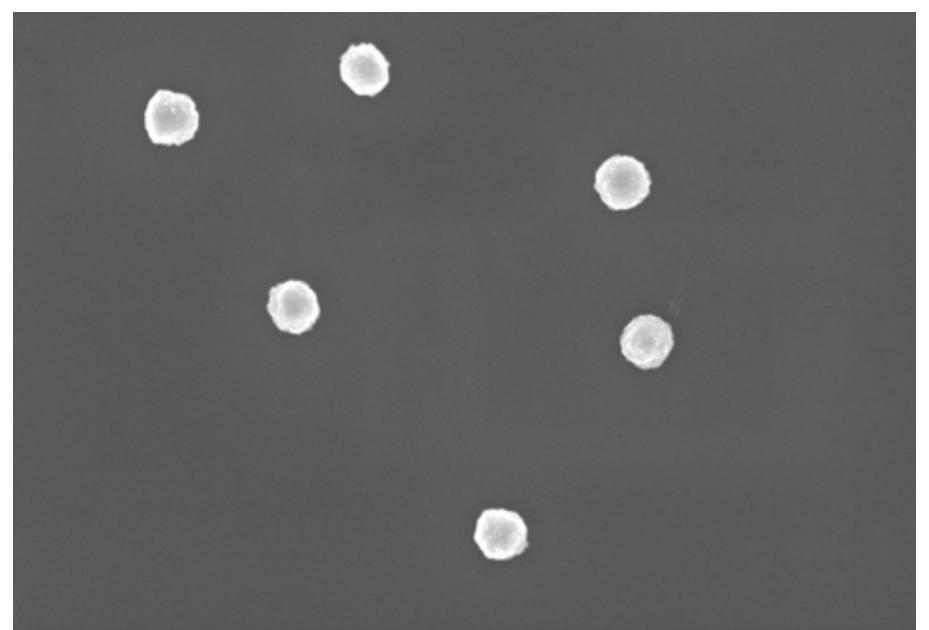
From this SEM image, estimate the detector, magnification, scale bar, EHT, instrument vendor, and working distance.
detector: InLens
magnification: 25 K X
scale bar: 200 nm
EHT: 5 kV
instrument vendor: Zeiss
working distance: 9.1 mm